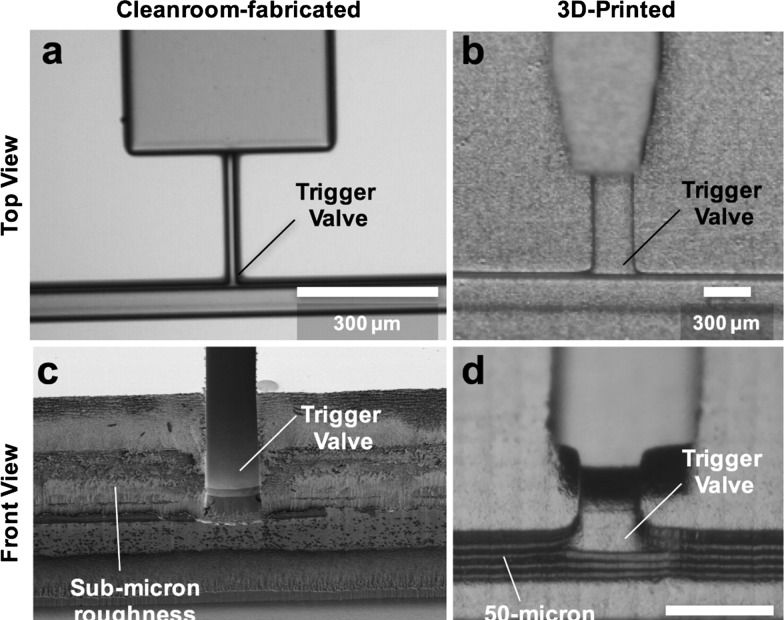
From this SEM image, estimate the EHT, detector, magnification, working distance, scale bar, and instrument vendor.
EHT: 1 kV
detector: SE(UL)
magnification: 0.6 K X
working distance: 20.1 mm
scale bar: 50000 nm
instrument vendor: Hitachi